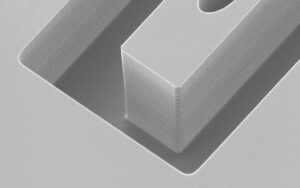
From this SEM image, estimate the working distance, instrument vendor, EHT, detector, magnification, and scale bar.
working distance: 11.9 mm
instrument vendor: Zeiss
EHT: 3 kV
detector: SESI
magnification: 3.59 K X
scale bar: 2000 nm